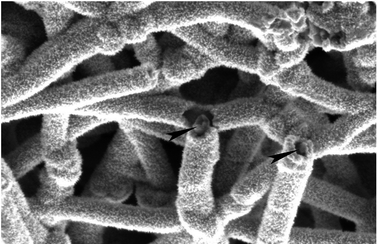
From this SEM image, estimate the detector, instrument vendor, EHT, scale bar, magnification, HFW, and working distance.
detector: T2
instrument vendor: FEI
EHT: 2 kV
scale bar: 1000 nm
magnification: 150 K X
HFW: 2.76 µm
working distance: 3 mm